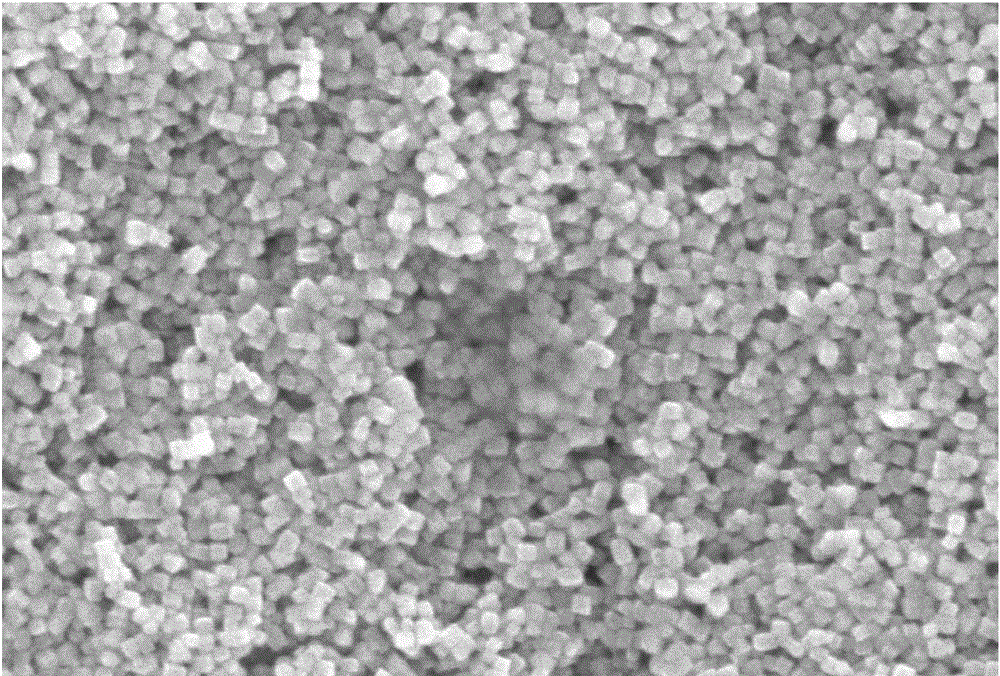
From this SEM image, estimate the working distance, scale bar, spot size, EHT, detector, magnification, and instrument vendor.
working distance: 10 mm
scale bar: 500 nm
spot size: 15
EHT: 10 kV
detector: SEI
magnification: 30 K X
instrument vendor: JEOL